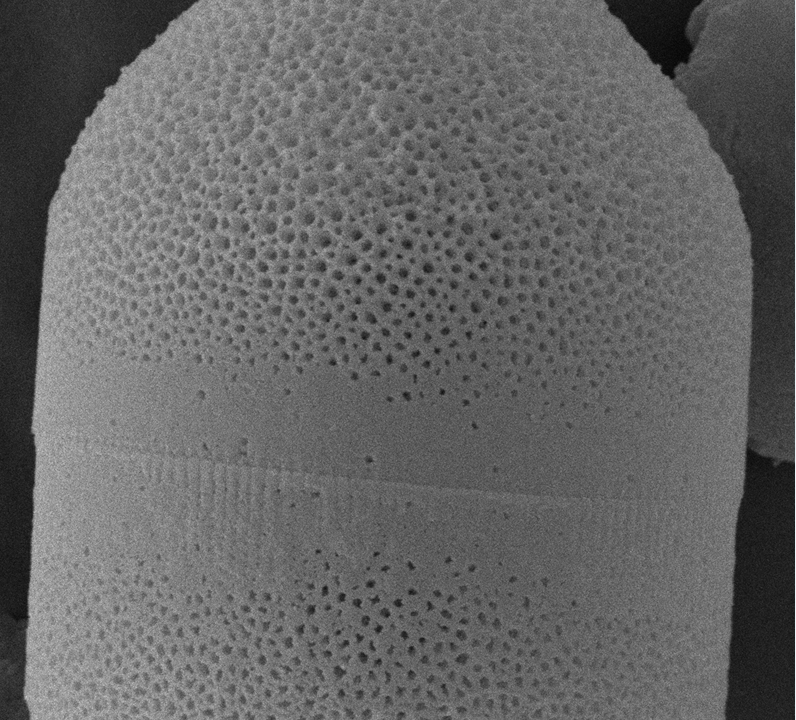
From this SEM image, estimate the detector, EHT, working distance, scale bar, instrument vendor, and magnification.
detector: SE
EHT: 15 kV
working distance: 8 mm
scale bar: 2000 nm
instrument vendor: other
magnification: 20 K X